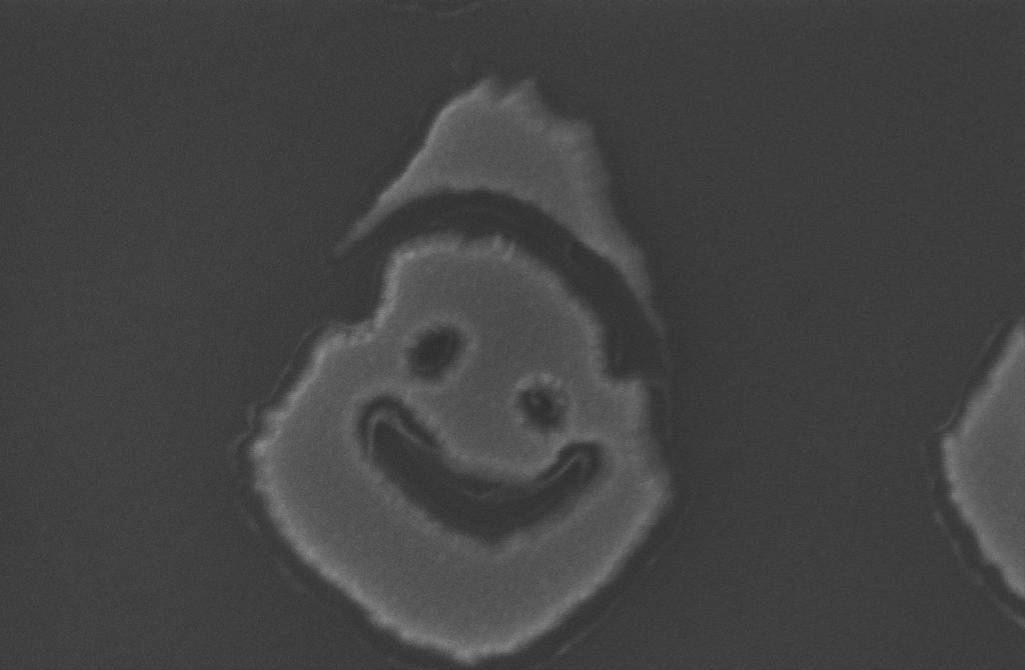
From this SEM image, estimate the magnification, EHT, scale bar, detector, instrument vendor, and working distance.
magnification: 279.9 K X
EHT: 5 kV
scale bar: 100 nm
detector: InLens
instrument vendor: Zeiss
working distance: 6 mm